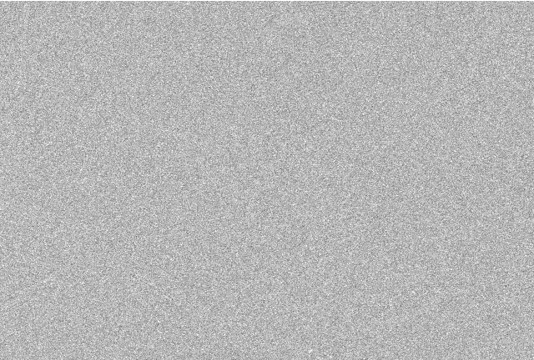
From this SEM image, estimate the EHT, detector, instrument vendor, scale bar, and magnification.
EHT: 15 kV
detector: SEI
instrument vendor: JEOL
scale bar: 5000 nm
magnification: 5 K X